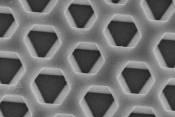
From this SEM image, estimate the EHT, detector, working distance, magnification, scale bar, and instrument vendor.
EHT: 5 kV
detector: SE(M)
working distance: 9.2 mm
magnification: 30 K X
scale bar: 1000 nm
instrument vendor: Hitachi